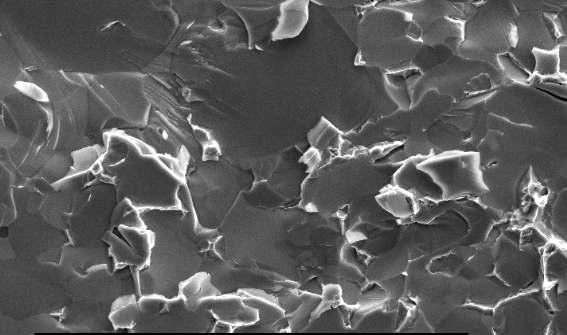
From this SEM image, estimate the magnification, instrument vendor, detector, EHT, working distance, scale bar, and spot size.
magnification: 10 K X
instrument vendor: FEI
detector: SE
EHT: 10 kV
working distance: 5 mm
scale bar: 5000 nm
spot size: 3